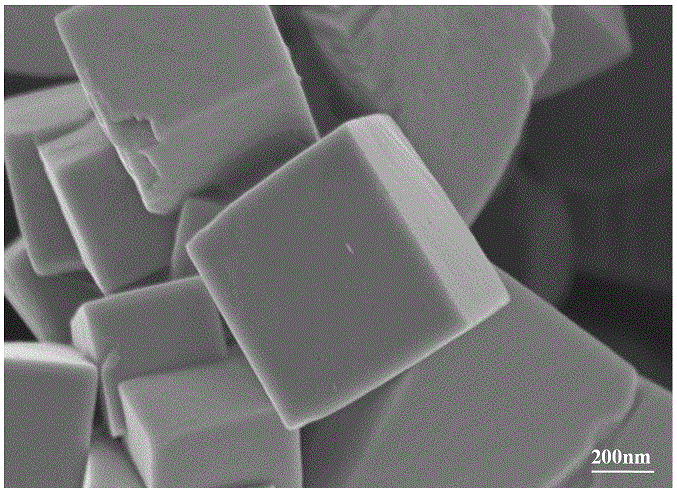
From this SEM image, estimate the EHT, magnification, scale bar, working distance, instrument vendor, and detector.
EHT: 3 kV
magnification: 50 K X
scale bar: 100 nm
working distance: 2.8 mm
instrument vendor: Zeiss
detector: InLens